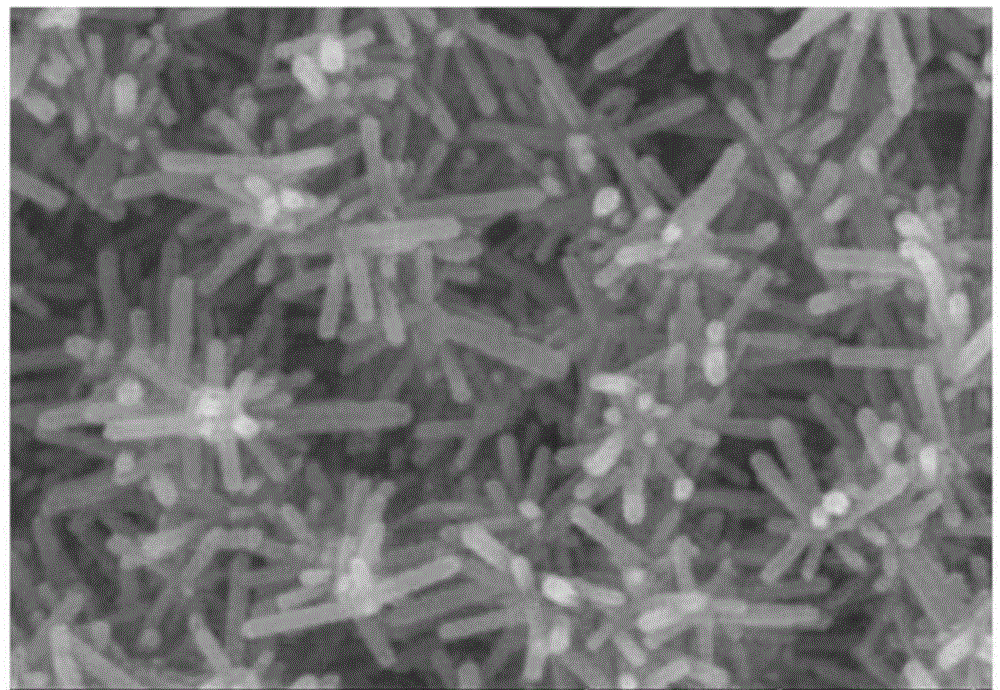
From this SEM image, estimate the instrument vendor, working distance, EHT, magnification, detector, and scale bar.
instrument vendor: Hitachi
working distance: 10.9 mm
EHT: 15 kV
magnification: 100 K X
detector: SE(UL)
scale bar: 500 nm